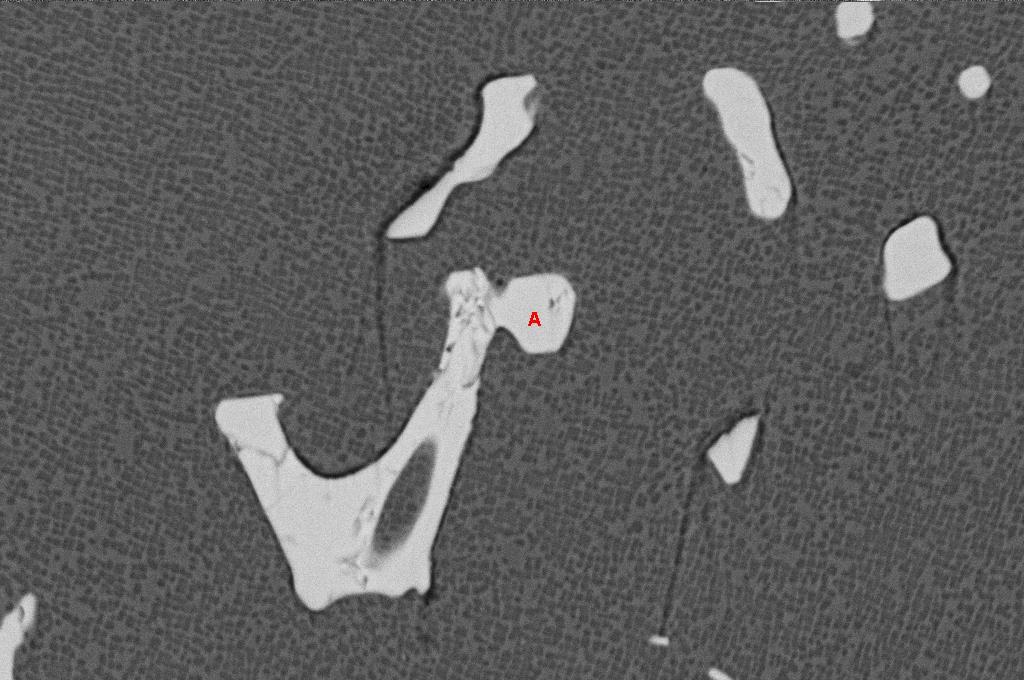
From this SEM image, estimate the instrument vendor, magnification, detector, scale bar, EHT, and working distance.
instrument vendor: Zeiss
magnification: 5 K X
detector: QBSD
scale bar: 10000 nm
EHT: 20 kV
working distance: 16 mm